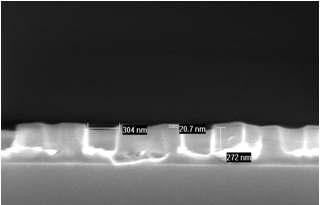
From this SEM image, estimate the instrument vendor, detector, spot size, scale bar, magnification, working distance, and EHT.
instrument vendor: FEI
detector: TLD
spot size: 3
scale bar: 500 nm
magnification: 100 K X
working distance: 5 mm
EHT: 10 kV